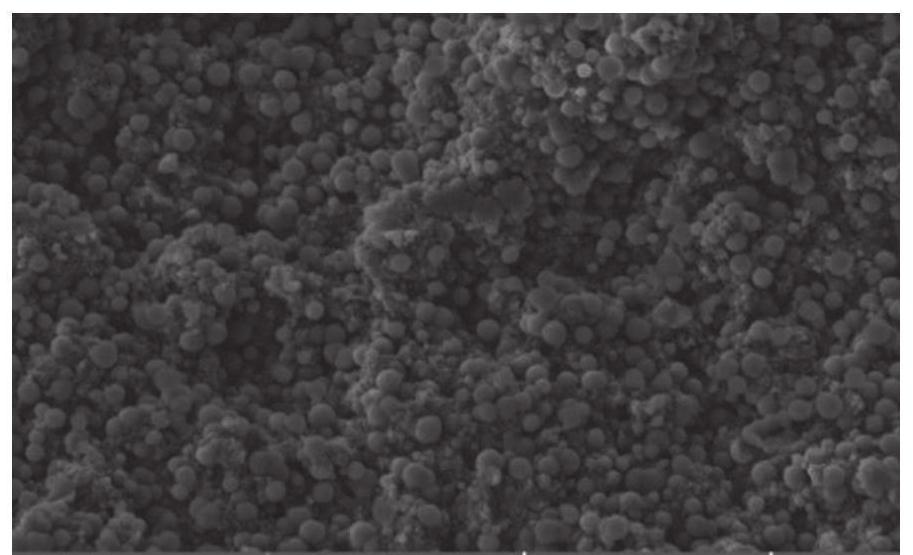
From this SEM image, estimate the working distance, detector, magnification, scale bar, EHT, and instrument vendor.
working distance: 9.93 mm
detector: SE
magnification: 10 K X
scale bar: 5000 nm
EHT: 5 kV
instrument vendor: Tescan